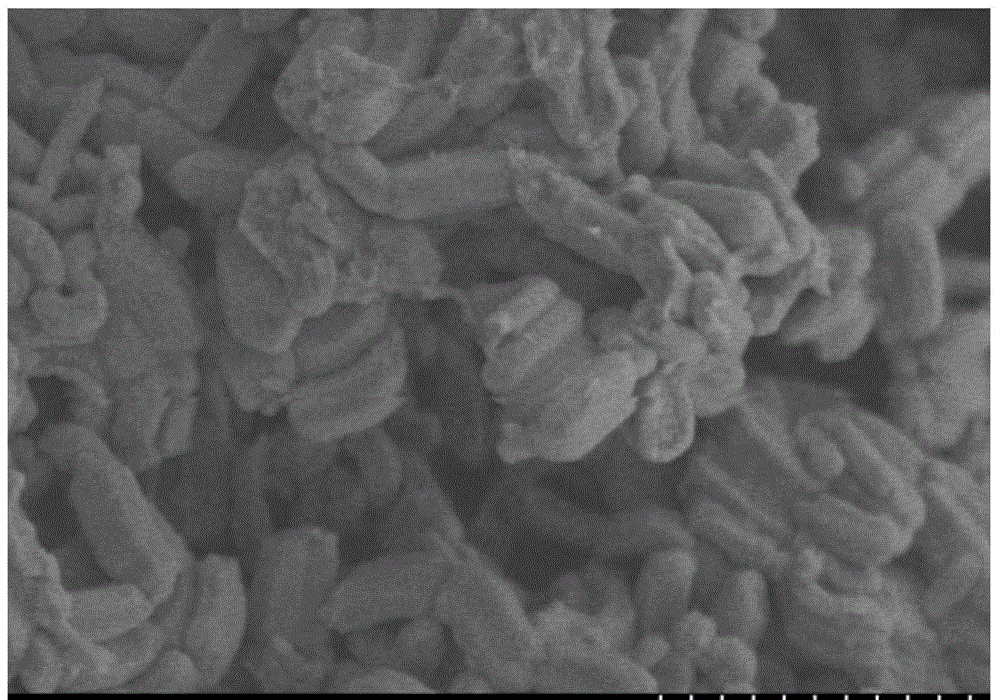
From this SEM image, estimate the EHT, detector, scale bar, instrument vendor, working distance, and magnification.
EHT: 2 kV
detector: SE(M)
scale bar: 2000 nm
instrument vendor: Hitachi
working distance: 7.4 mm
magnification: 20 K X